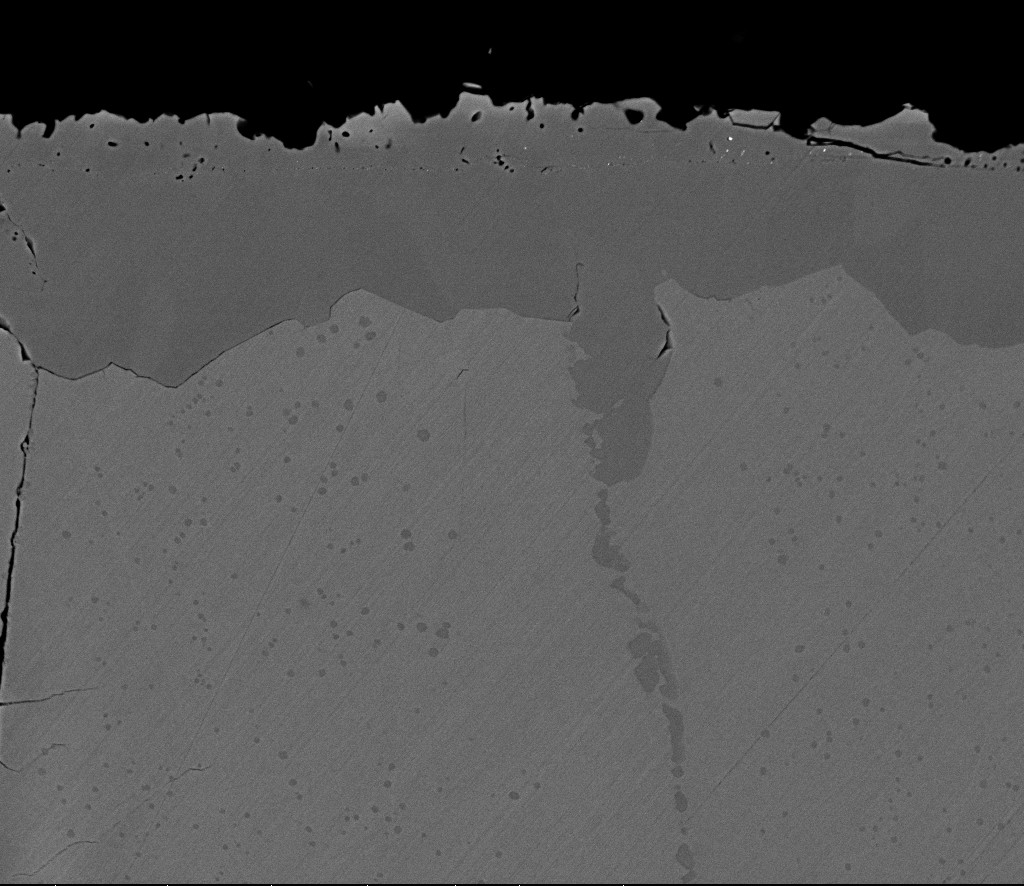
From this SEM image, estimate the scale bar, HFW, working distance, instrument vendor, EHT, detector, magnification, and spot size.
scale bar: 50000 nm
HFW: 149 µm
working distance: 5.9 mm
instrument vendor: FEI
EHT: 18 kV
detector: BSED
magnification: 2 K X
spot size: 4